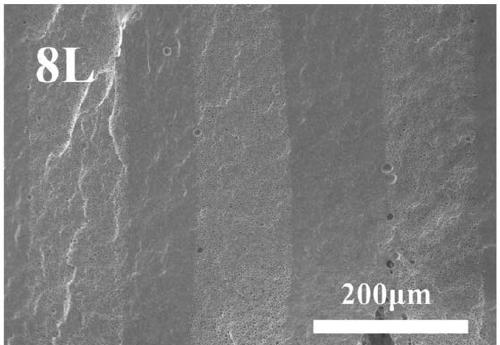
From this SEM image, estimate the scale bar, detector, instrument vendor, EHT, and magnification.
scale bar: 200000 nm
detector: SED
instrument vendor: other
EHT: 10 kV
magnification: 0.2 K X